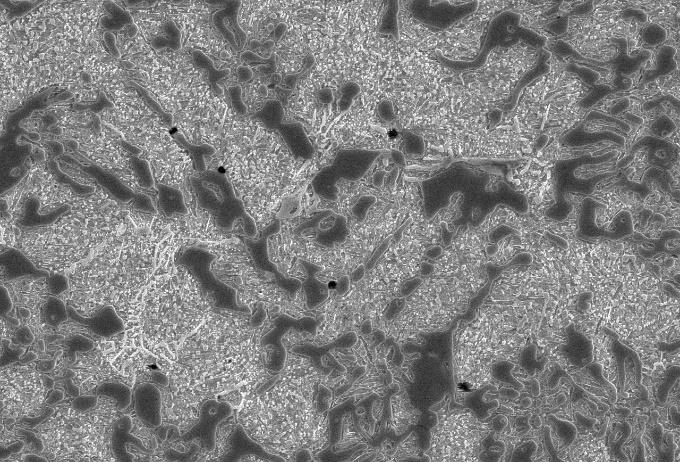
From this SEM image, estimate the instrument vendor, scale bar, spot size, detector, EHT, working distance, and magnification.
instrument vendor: JEOL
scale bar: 10000 nm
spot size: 50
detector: SEI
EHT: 20 kV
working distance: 12 mm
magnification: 1.5 K X